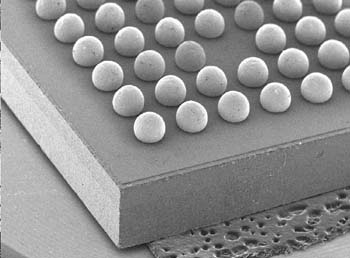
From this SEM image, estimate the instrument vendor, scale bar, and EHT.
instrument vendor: JEOL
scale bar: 1000000 nm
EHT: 20 kV